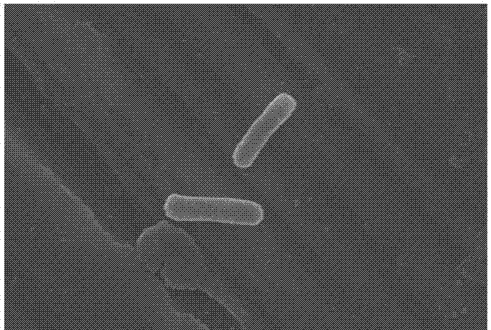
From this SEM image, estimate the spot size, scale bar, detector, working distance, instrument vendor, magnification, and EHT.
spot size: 30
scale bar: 1000 nm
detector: SEI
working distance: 14 mm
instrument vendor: JEOL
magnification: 10 K X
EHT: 20 kV